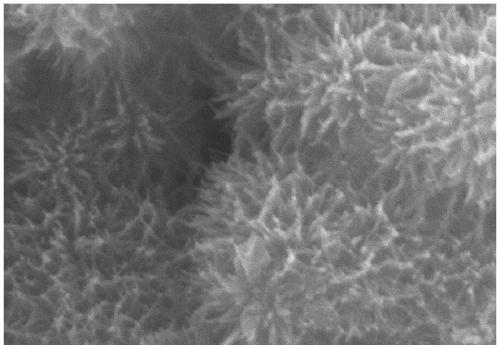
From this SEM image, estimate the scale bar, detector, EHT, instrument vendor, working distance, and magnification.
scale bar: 1000 nm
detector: SE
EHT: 20 kV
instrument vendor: Hitachi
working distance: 9.8 mm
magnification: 30 K X